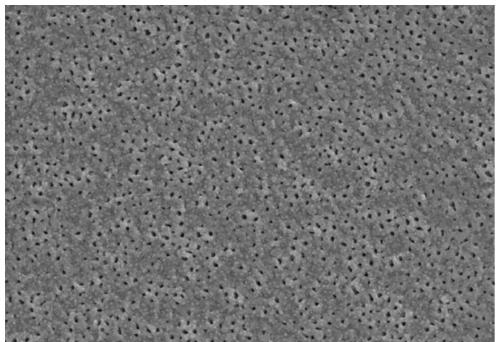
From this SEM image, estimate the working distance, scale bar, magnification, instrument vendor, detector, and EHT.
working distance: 10 mm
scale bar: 10000 nm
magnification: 5 K X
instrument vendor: Hitachi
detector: SE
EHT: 15 kV